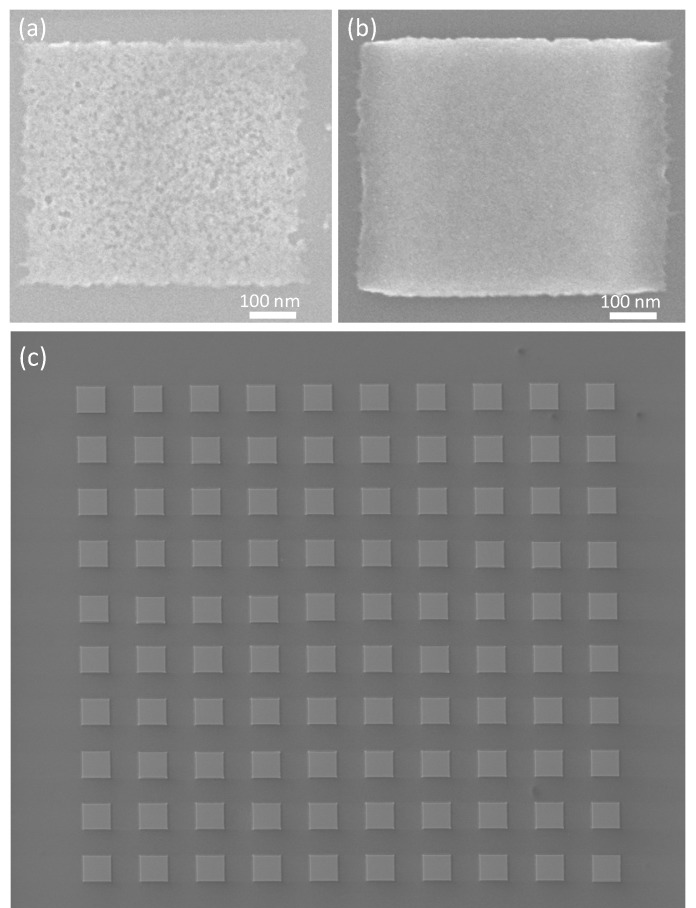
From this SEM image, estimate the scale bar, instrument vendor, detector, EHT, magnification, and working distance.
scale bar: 50000 nm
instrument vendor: FEI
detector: CDEM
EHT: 5 kV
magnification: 1.25 K X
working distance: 5.4 mm